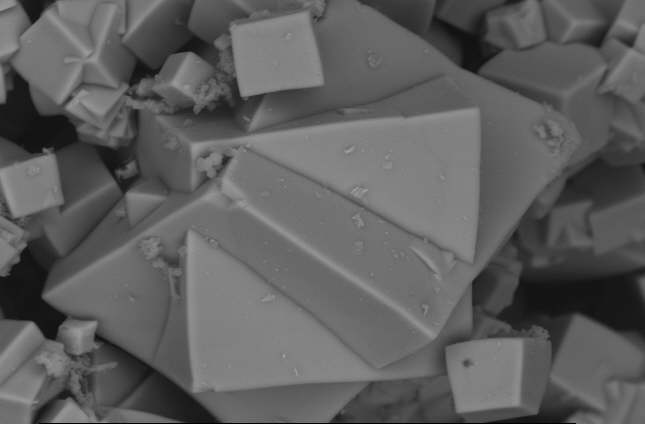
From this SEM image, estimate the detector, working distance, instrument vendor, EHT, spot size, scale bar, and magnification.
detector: BSE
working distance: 11.1 mm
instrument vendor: FEI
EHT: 20 kV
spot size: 5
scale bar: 10000 nm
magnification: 2 K X